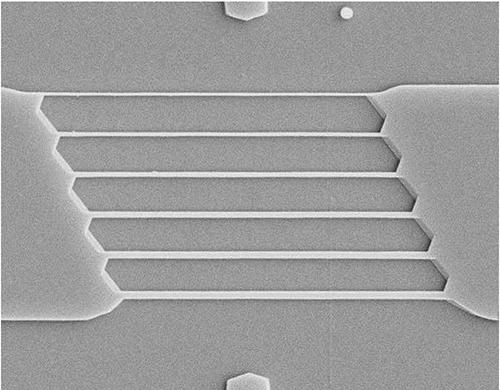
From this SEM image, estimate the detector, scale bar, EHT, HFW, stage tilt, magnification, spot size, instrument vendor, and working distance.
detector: CDM-E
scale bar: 2000 nm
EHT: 10 kV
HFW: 15.2 µm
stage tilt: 0°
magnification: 10 K X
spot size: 3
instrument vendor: FEI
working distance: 4.921 mm